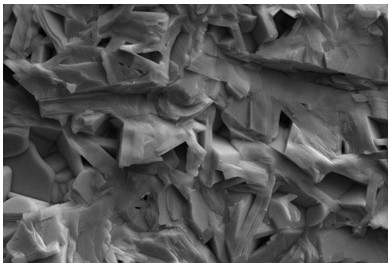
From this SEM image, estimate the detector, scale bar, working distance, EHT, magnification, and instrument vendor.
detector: SE2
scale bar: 1000 nm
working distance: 8.7 mm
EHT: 15 kV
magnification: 4 K X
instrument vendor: Zeiss